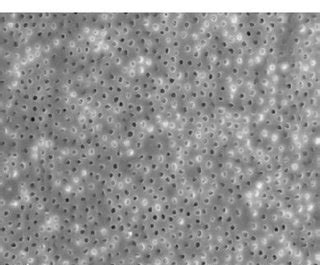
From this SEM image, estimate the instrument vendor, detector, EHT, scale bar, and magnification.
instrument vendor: Zeiss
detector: SE1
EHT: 20 kV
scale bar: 20000 nm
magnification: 2 K X